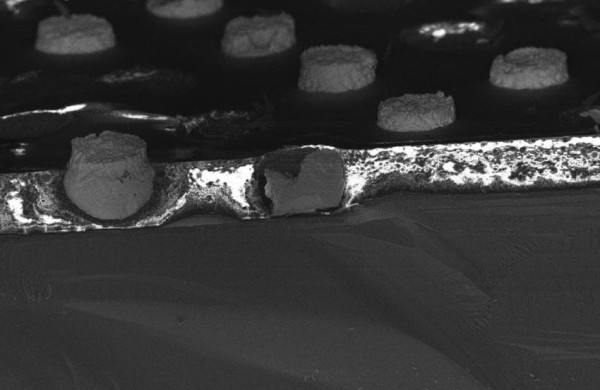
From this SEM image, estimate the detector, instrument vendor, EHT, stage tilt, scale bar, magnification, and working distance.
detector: InLens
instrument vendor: Zeiss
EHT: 15 kV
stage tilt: -15°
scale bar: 100000 nm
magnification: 0.283 K X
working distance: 4 mm